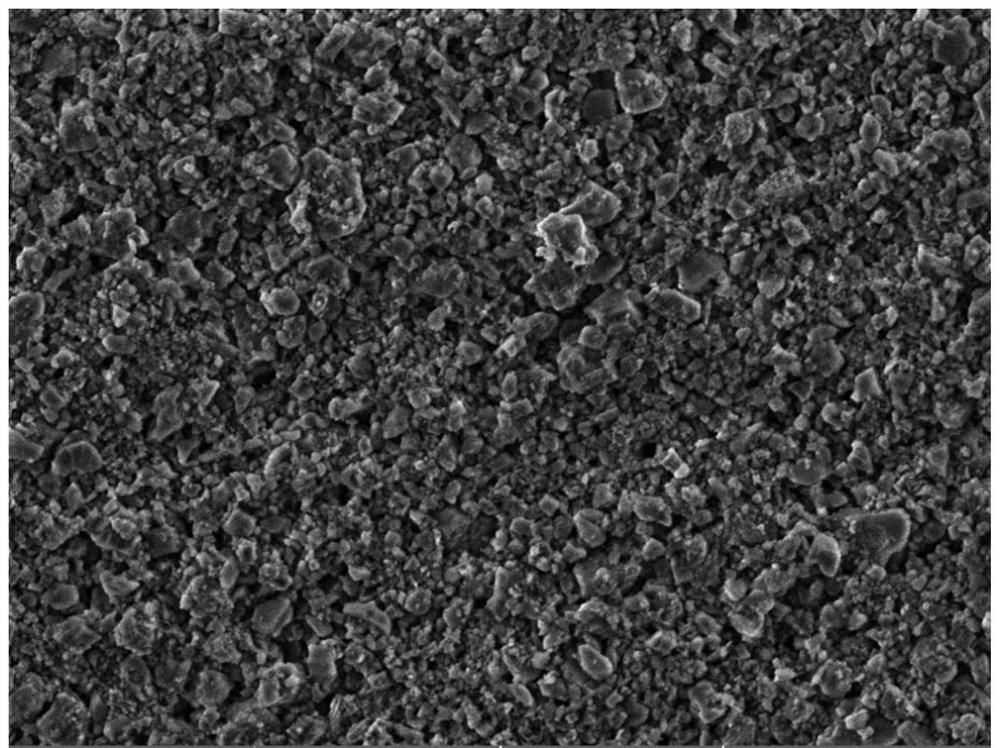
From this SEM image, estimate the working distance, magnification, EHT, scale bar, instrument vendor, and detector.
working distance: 10 mm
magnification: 6.26 K X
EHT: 5 kV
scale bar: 10000 nm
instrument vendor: Tescan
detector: SE